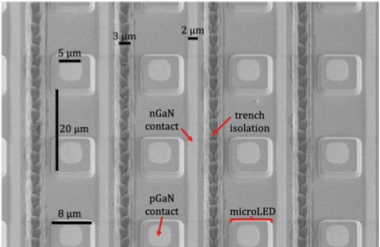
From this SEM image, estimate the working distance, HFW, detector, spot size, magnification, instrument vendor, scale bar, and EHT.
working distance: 5.4 mm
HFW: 84.7 µm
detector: ETD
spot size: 3.5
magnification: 1.5 K X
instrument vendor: FEI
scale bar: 10000 nm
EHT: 5 kV